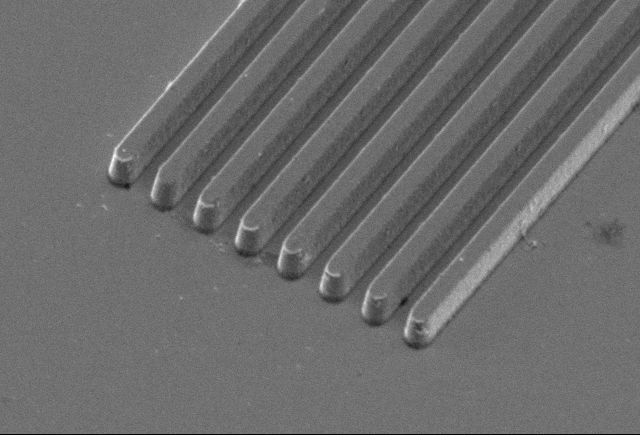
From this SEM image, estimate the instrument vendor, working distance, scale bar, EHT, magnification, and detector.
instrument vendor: Hitachi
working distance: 11.4 mm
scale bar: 10000 nm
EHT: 5 kV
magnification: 5 K X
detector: SE